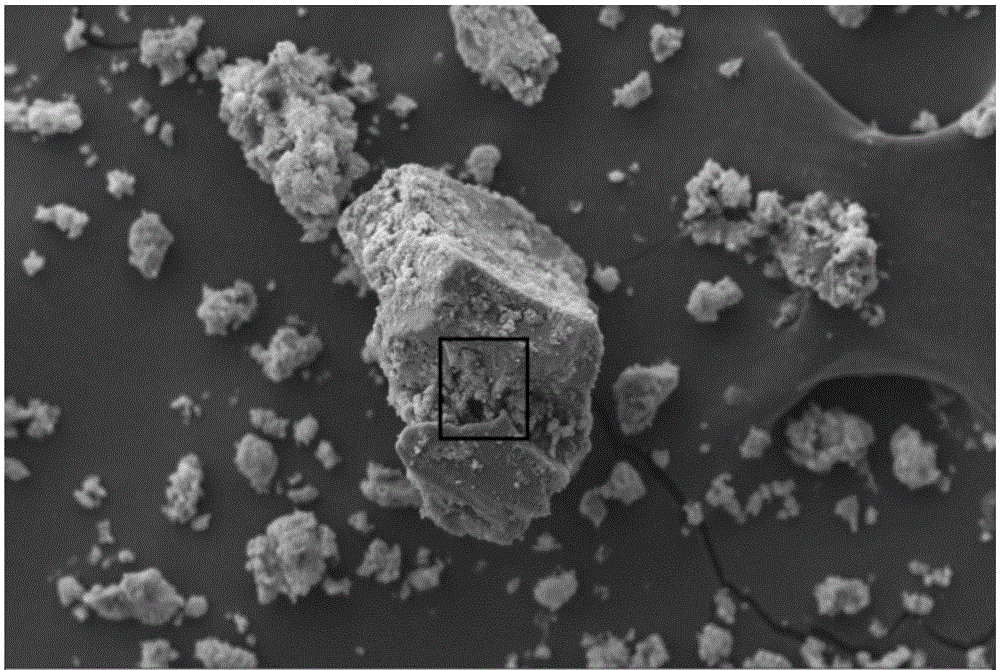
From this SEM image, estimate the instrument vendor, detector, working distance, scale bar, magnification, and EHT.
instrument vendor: Zeiss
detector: SE1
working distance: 8 mm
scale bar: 10000 nm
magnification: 1.5 K X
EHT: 10 kV